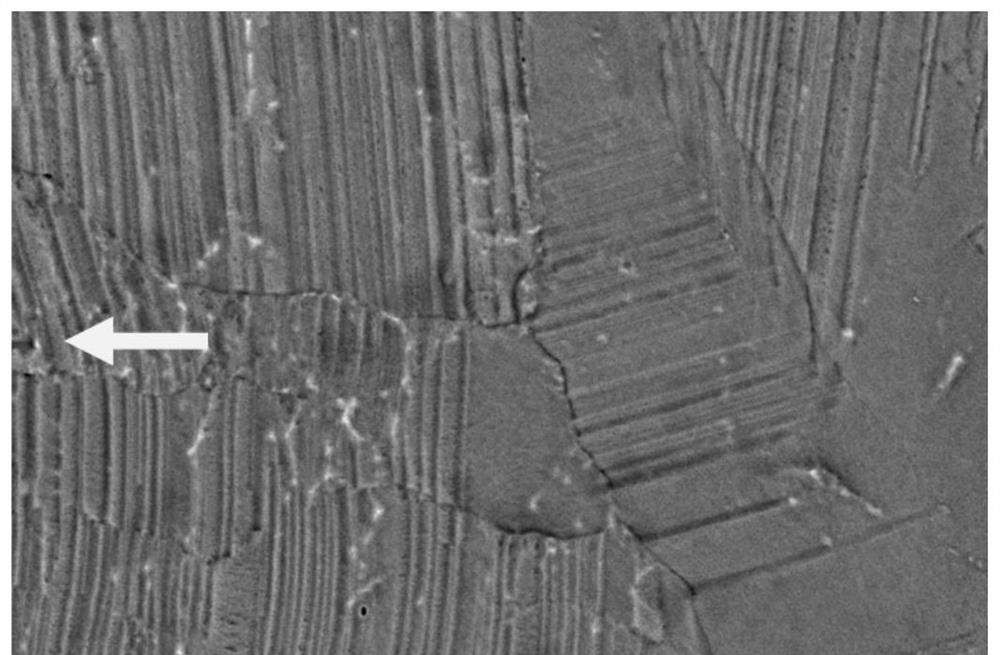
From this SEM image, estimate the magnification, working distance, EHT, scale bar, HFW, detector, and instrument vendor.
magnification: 50 K X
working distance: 2.8 mm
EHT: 5 kV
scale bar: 4000 nm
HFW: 8.29 µm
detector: T1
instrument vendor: FEI